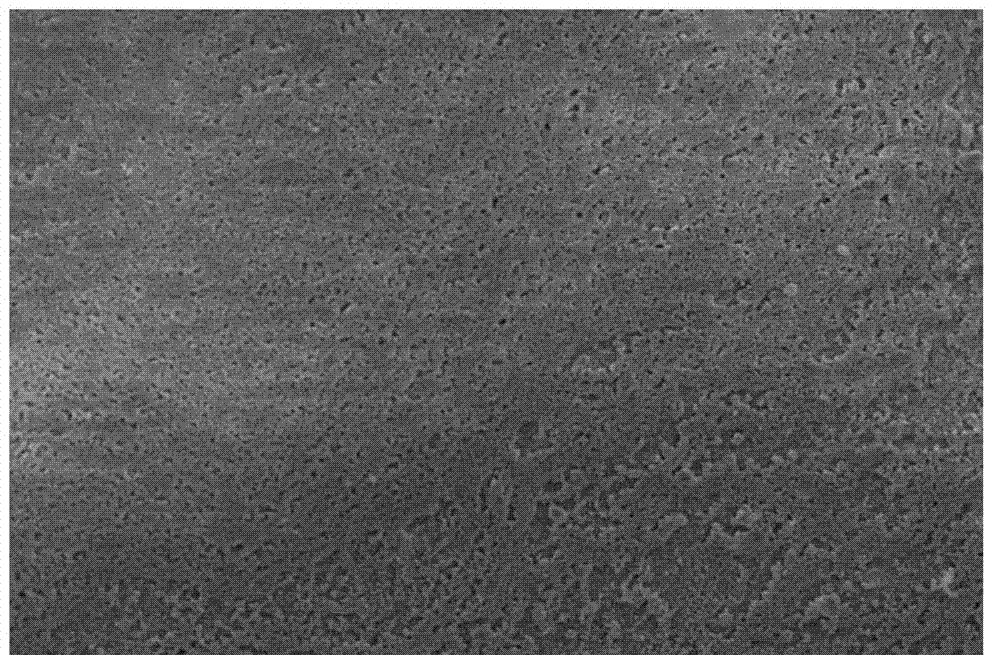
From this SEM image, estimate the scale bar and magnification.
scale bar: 50000 nm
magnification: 0.5 K X